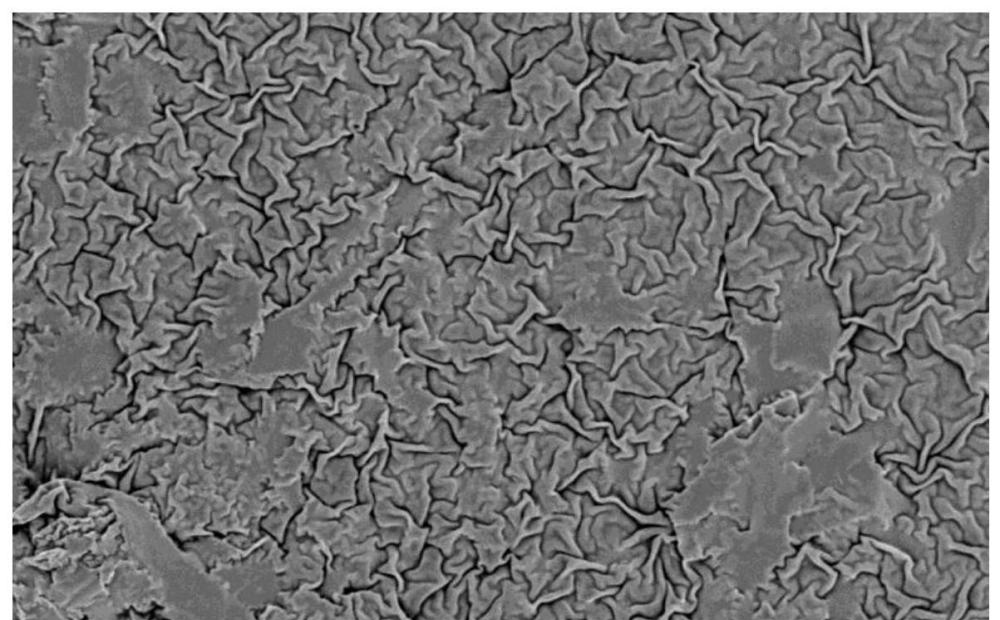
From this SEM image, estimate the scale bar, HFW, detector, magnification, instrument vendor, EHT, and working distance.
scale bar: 80000 nm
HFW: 259 µm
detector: BSD Full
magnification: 2 K X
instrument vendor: other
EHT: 10 kV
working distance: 7.54 mm